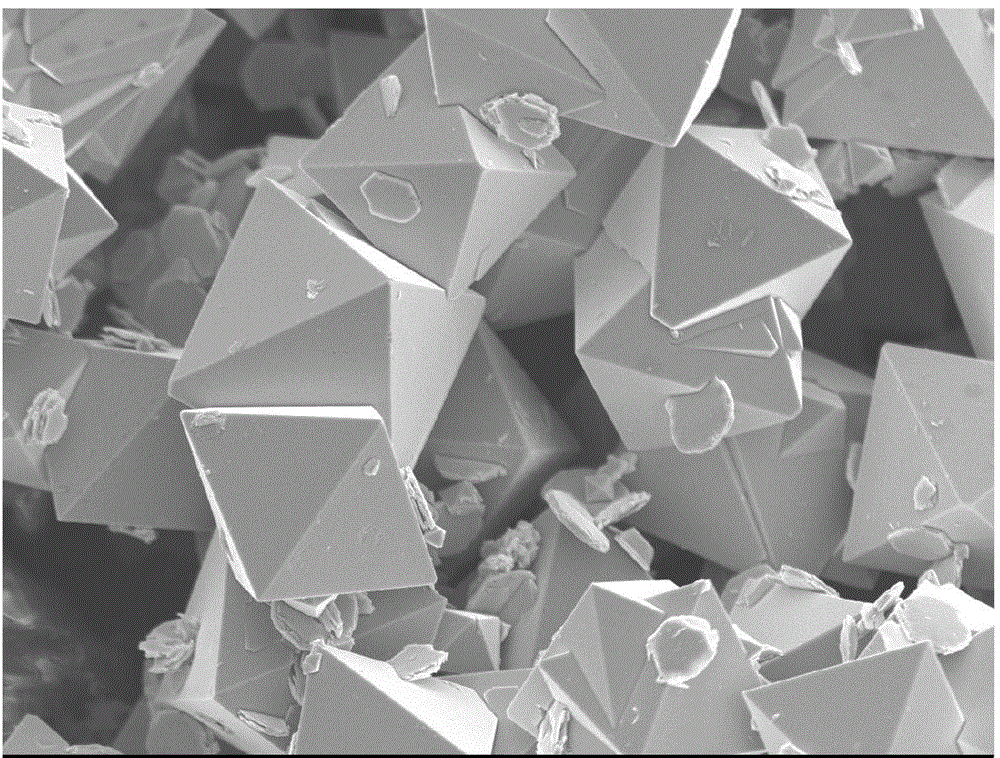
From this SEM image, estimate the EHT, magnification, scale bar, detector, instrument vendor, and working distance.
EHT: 5 kV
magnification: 5 K X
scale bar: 1000 nm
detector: SEI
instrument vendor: JEOL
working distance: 6.6 mm